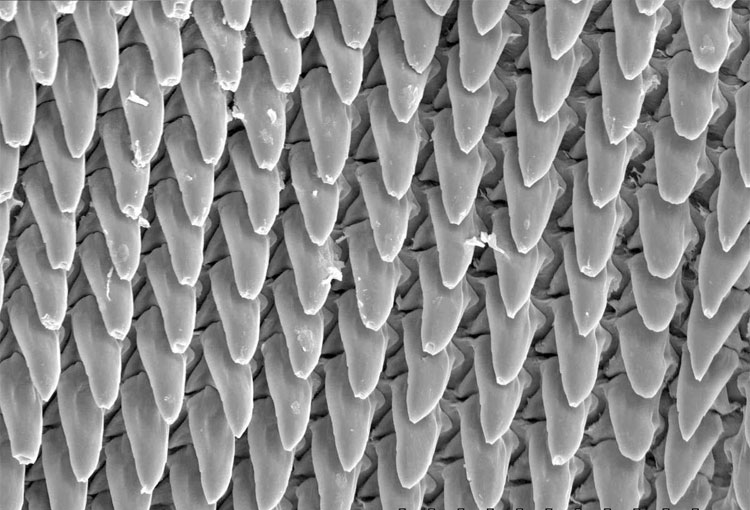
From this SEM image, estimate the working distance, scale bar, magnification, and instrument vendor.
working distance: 16.4 mm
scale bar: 100000 nm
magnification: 0.5 K X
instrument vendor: Hitachi